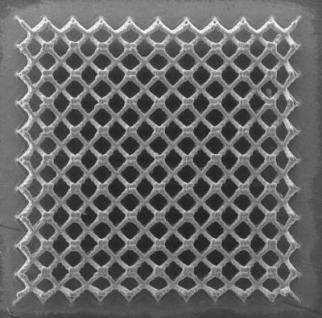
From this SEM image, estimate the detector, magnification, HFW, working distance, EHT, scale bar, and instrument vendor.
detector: SE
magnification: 0.092 K X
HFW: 2260 µm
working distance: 12.62 mm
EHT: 20 kV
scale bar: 500000 nm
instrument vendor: Tescan